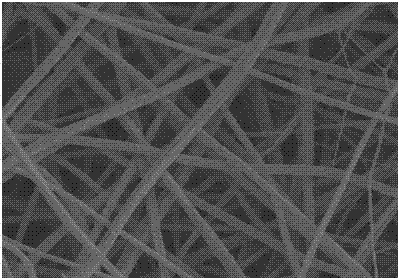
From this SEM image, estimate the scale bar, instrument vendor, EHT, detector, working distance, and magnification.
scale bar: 5000 nm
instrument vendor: Hitachi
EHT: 5 kV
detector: SE(M)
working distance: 8.5 mm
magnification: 10 K X